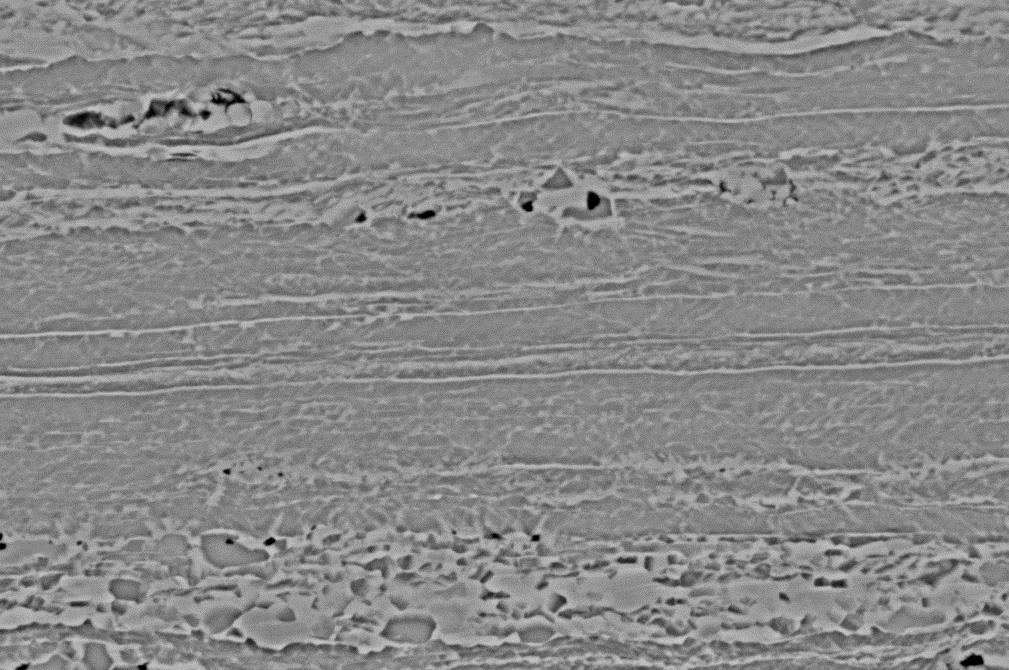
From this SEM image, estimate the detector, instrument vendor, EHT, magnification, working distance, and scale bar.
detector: QBSD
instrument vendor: Zeiss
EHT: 20 kV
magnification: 5 K X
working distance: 10 mm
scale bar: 2000 nm